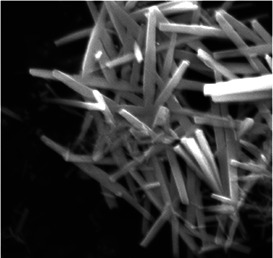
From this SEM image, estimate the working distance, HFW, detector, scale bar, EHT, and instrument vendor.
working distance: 32.34 mm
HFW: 5.54 µm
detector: SE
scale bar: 1000 nm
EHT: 10 kV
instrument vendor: Tescan